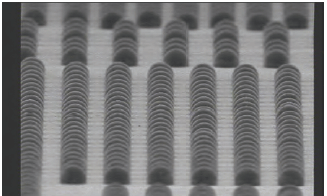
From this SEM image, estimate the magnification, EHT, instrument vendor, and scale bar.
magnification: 0.346 K X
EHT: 10 kV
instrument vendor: JEOL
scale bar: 86800 nm